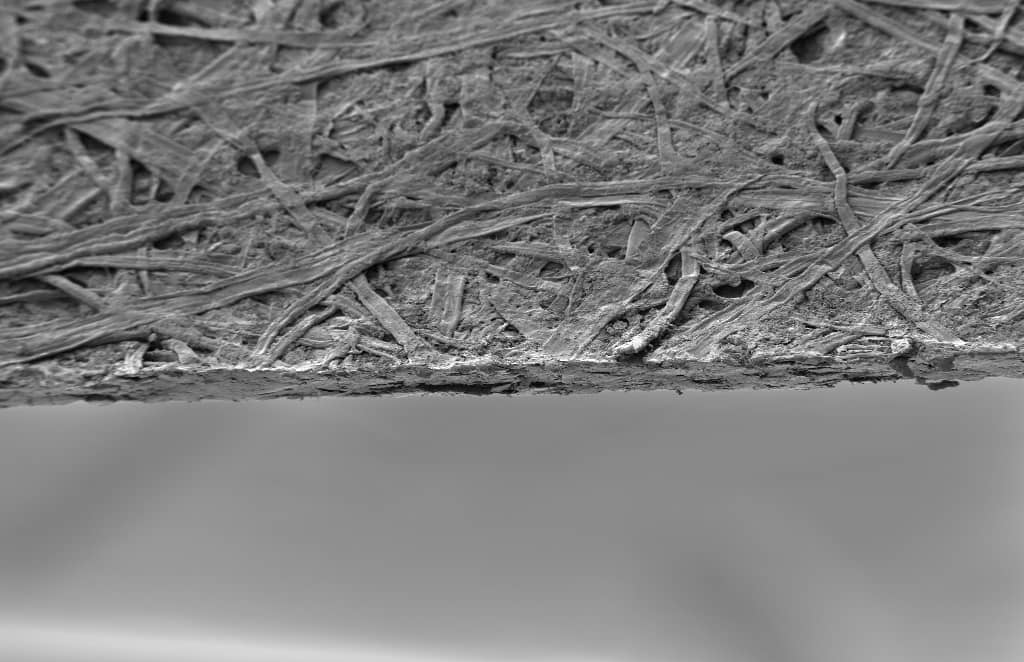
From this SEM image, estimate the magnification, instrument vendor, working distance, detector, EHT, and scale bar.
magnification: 0.1 K X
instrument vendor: Zeiss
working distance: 14.5 mm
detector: SE2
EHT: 3 kV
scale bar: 100000 nm